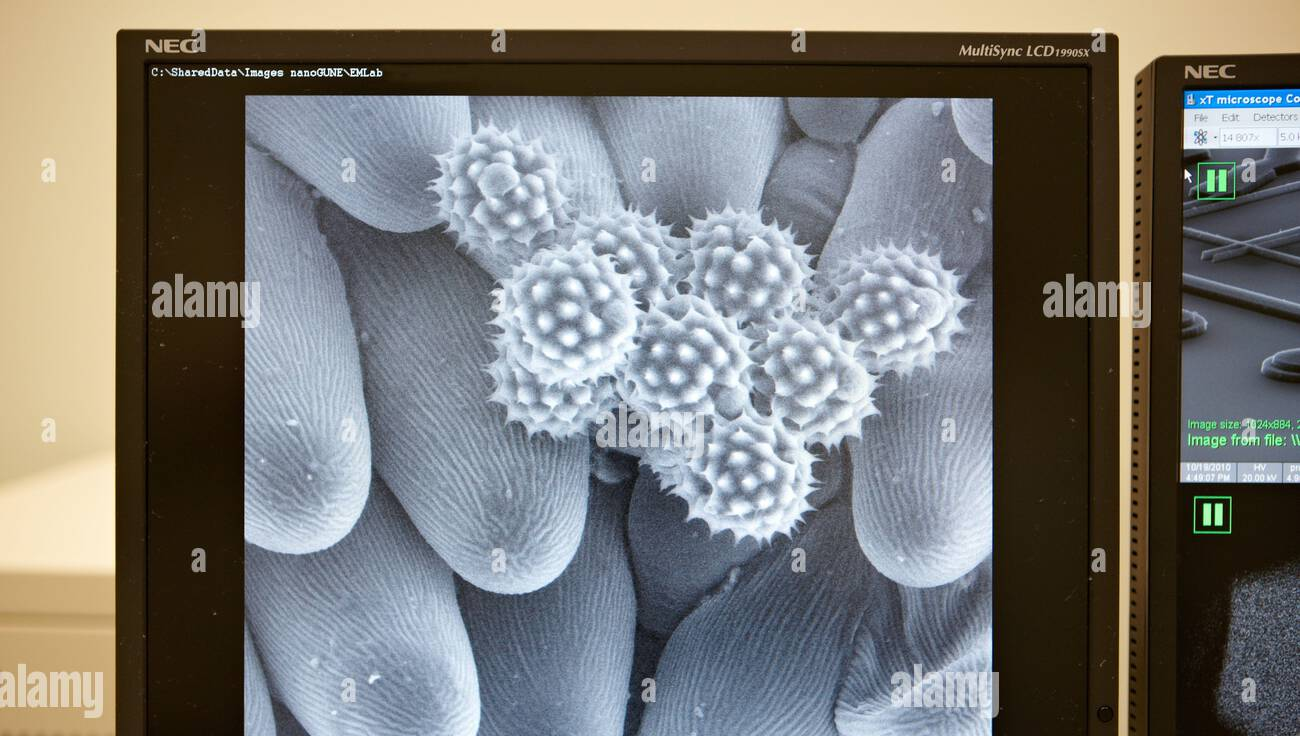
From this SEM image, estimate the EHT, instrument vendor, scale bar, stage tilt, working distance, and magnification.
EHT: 10 kV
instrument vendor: FEI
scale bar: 30000 nm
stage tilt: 0°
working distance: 8.7 mm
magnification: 3 K X